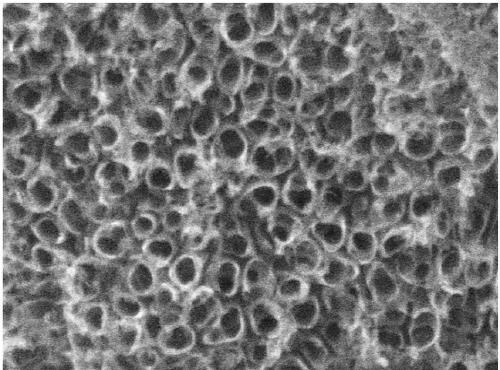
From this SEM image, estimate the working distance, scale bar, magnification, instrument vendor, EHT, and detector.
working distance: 5.5 mm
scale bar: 100 nm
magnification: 150 K X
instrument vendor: JEOL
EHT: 10 kV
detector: SEI